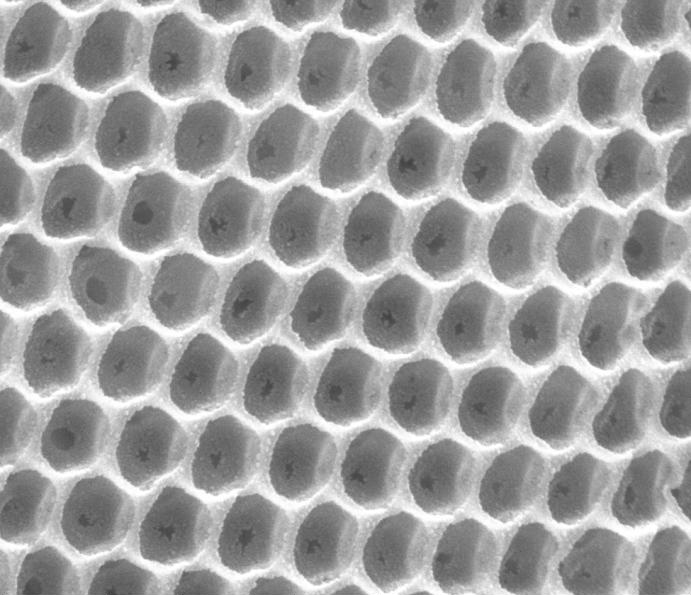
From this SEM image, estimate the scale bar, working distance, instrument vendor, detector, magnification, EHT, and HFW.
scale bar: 500 nm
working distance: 12.1 mm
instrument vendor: FEI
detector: ETD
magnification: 100 K X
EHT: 20 kV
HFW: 2.56 µm